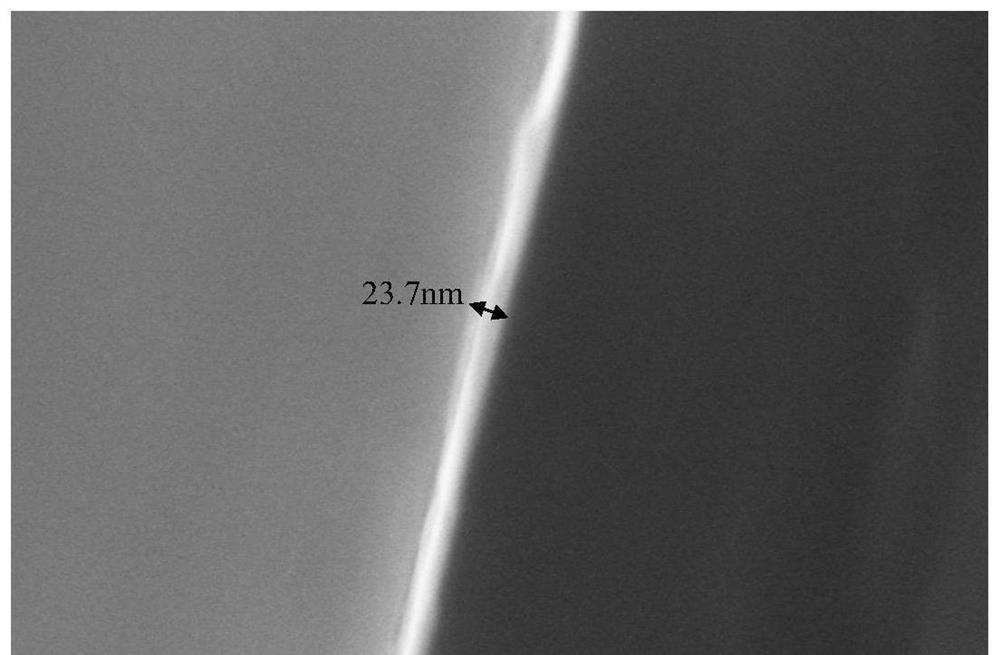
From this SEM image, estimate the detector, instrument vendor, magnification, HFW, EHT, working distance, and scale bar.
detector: TLD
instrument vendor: FEI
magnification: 250 K X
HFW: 0.829 µm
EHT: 10 kV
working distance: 3.4 mm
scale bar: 200 nm